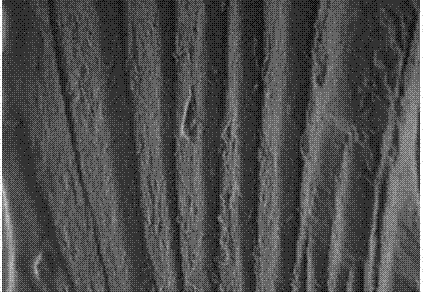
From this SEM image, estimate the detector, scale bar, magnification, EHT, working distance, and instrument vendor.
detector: SE(U)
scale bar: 2000 nm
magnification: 20 K X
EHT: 1 kV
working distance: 8.1 mm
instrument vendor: Hitachi